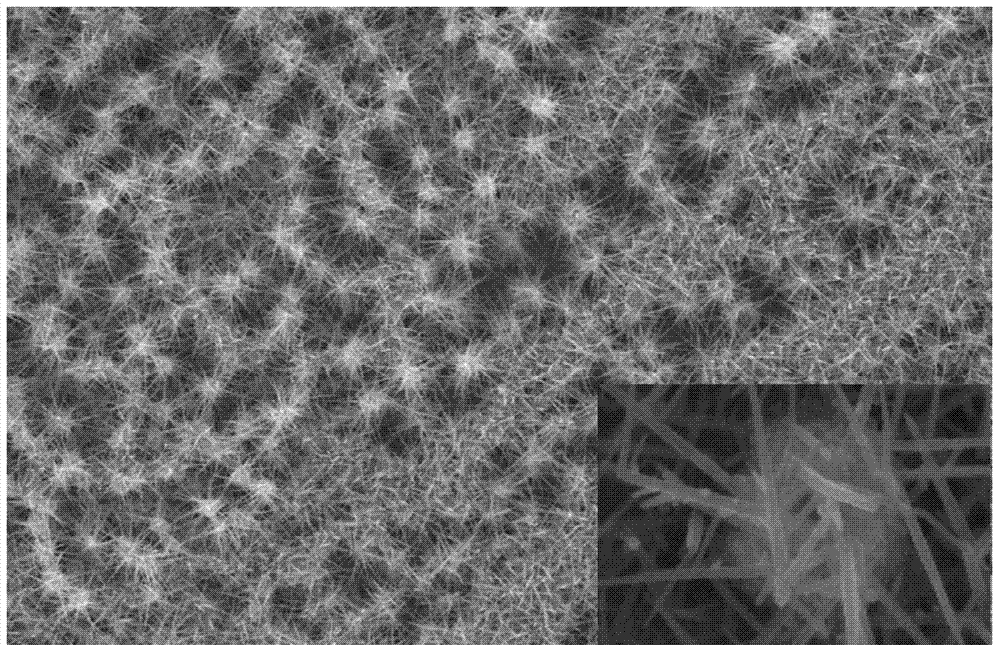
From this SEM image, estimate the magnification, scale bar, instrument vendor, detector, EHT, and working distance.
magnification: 6 K X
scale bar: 5000 nm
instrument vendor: Hitachi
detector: SE(UL)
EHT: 10 kV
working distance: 9.4 mm